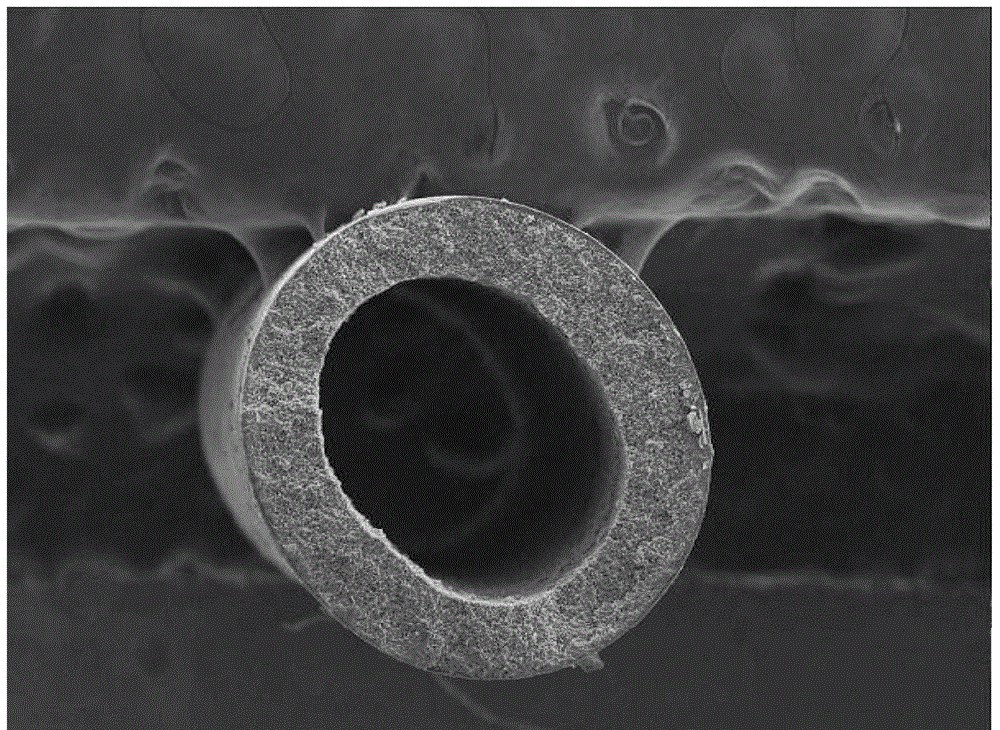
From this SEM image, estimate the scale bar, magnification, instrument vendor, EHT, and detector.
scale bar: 200000 nm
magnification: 0.05 K X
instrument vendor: other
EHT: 20 kV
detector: SE